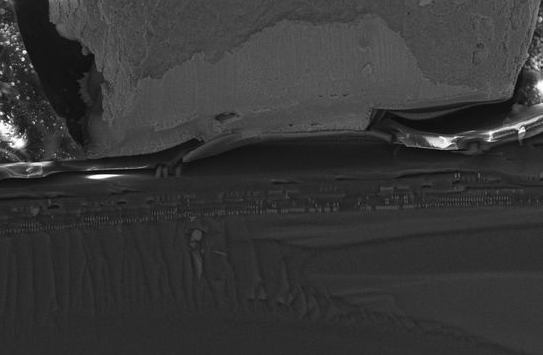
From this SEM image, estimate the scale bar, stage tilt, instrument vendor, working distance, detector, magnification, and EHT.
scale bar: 10000 nm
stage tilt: -15°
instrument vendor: Zeiss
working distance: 4 mm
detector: InLens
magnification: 2 K X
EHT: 15 kV